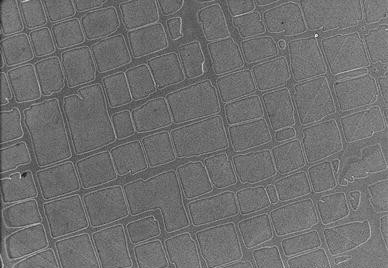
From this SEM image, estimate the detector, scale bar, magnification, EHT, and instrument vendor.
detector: SE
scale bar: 1500 nm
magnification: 20 K X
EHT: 200 kV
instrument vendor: Hitachi